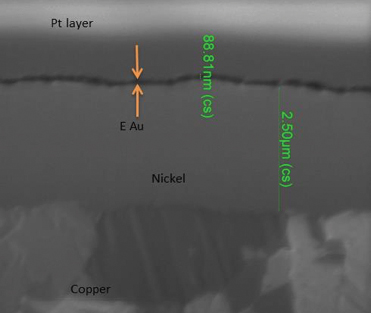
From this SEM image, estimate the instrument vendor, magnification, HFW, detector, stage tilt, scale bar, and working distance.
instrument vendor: FEI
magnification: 50 K X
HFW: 5.97 µm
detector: ETD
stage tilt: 0°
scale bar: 3000 nm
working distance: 30.3 mm